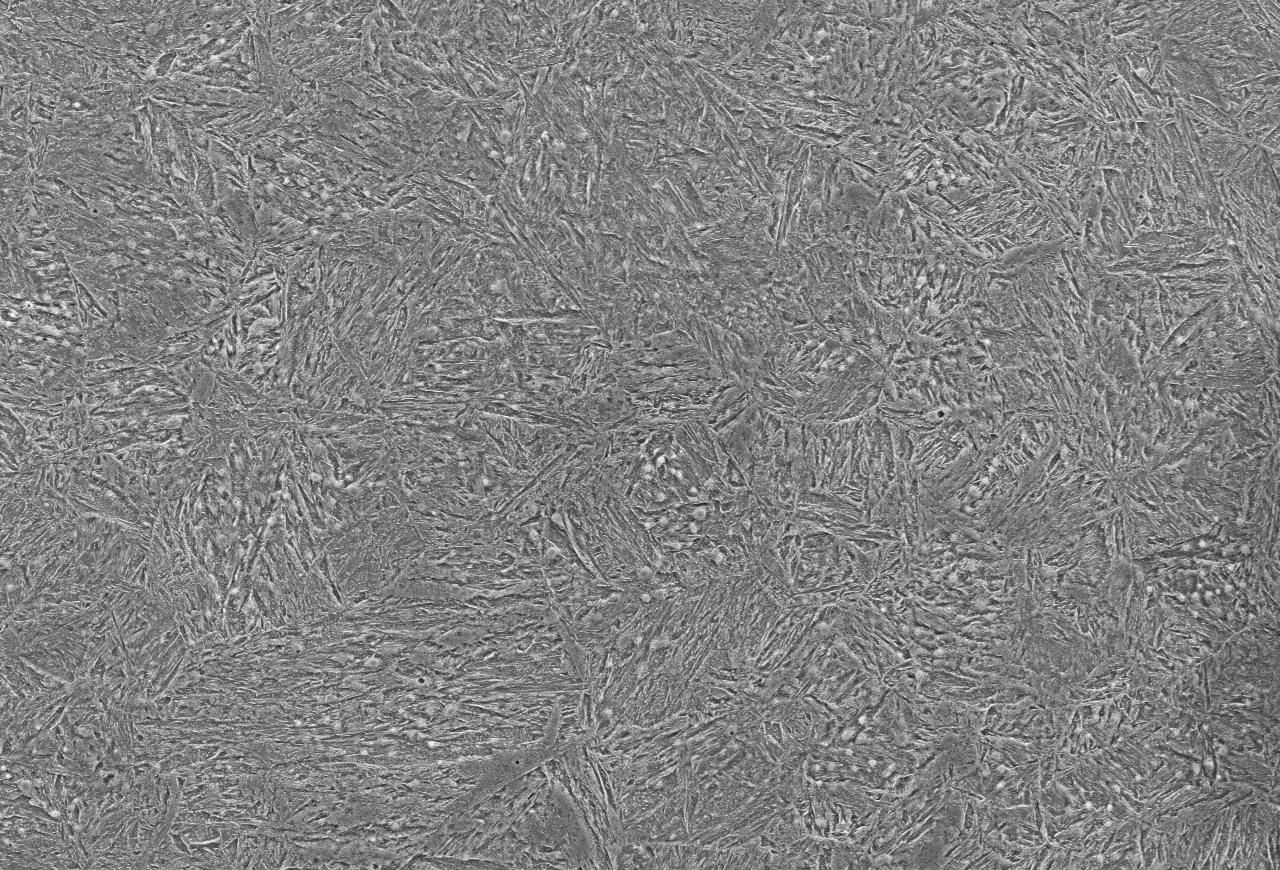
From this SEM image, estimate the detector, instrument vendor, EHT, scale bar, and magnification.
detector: SEI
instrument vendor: JEOL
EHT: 15 kV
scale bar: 20000 nm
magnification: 1 K X